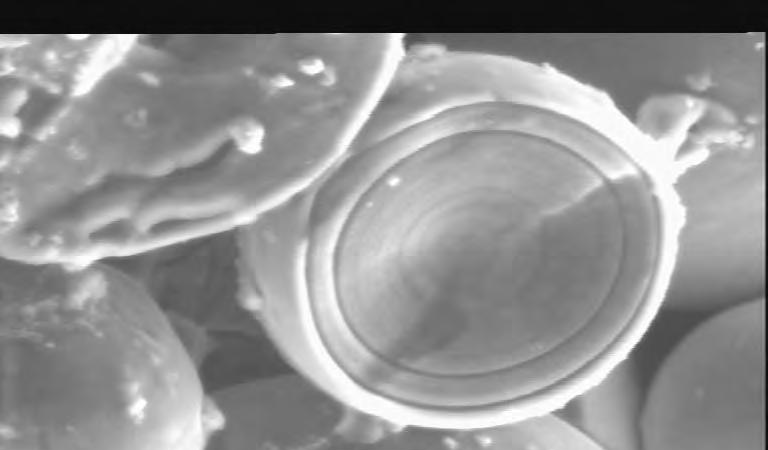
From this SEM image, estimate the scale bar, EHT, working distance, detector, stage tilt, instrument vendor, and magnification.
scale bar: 5000 nm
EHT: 10 kV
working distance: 8.1 mm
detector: ESD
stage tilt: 3.8°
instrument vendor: other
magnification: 2.6 K X